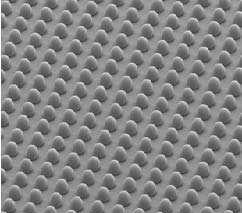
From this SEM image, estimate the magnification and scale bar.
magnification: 0.2 K X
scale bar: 200000 nm